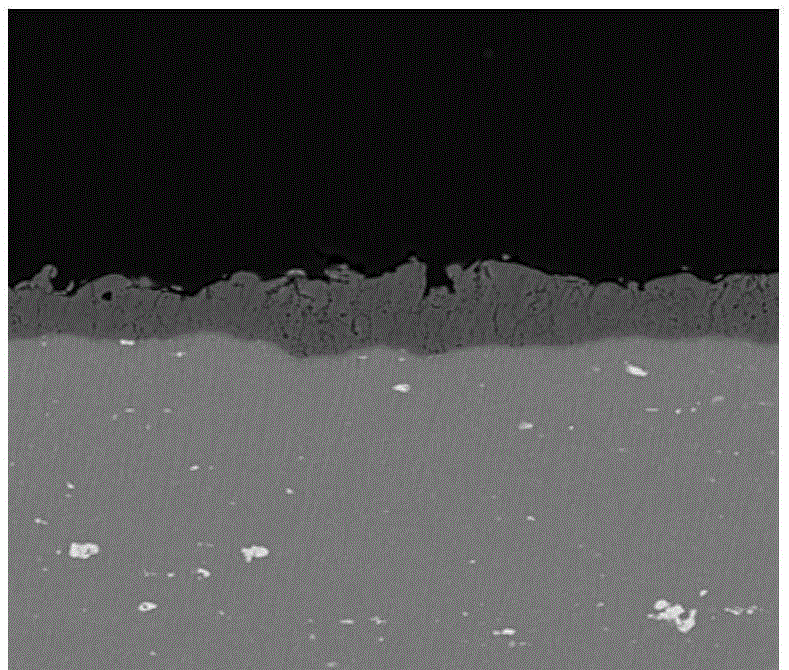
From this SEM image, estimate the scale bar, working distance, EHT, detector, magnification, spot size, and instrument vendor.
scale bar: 50000 nm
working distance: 10.8 mm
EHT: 18 kV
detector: BSED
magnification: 1 K X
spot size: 4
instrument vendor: FEI